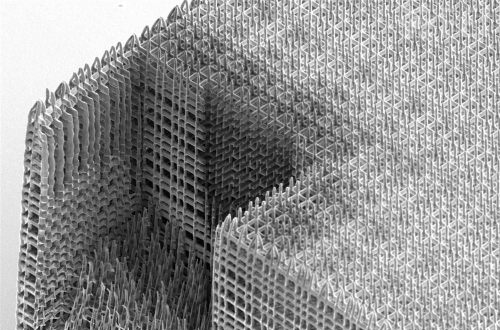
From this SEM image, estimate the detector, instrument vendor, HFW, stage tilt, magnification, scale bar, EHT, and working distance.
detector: ETD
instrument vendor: FEI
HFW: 59.2 µm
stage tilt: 52°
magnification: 3.5 K X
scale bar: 10000 nm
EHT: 2 kV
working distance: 3.7 mm